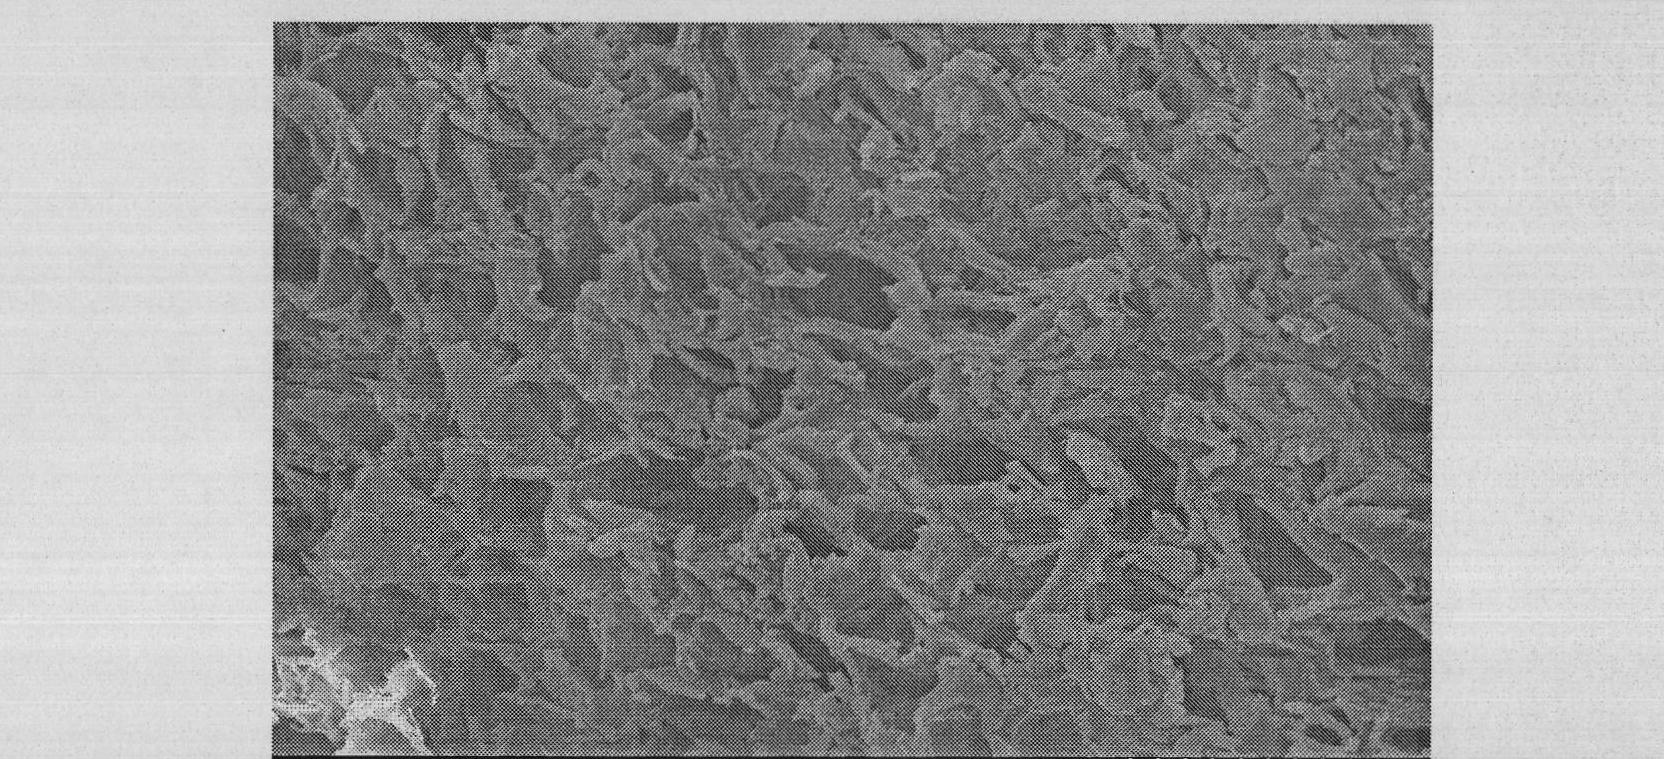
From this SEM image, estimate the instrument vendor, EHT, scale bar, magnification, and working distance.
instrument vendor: Hitachi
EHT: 20 kV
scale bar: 100000 nm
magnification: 0.3 K X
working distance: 11.2 mm